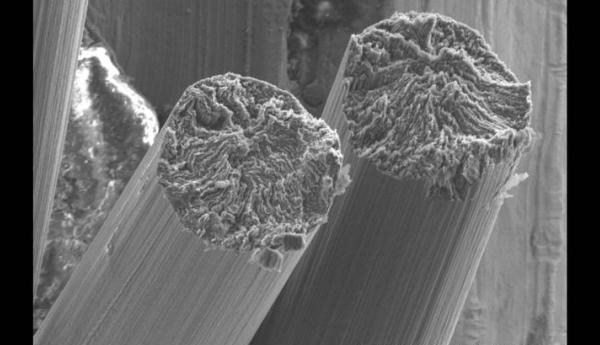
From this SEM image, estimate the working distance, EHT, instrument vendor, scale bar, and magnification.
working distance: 9 mm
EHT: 5 kV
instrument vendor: Hitachi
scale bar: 20000 nm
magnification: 2 K X